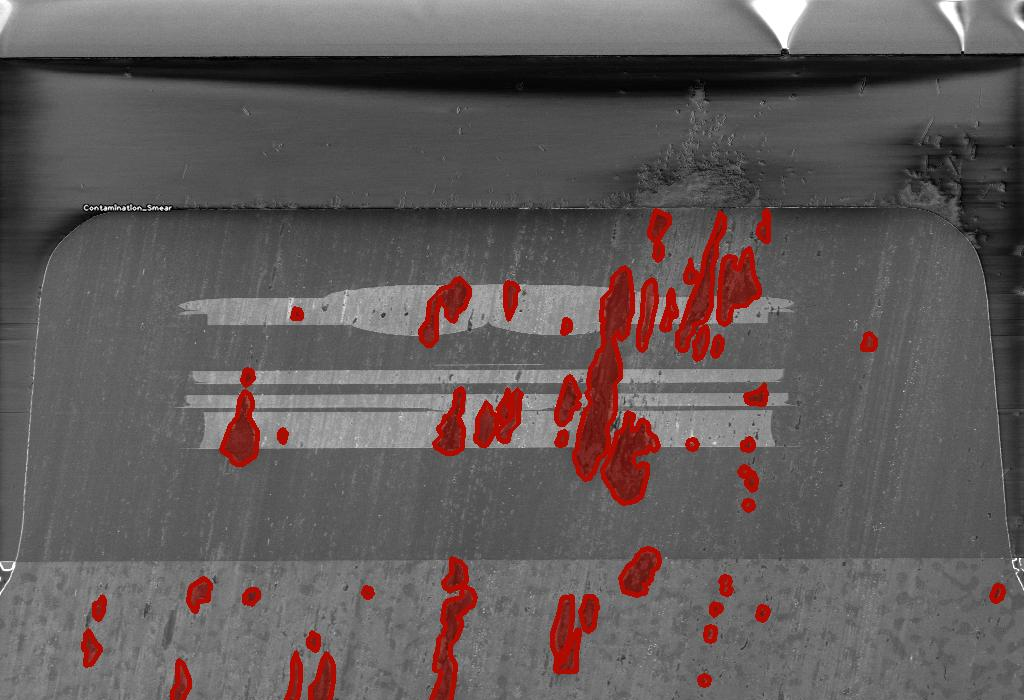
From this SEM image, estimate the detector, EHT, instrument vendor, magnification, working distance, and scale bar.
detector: InLens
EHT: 1 kV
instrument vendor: Zeiss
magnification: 2 K X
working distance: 2.6 mm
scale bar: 2000 nm